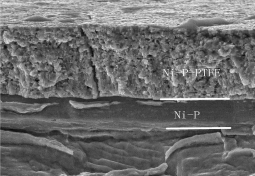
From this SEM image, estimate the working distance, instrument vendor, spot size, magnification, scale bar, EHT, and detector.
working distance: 8 mm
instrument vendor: FEI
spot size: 3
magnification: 25 K X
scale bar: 5000 nm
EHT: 10 kV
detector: ETD